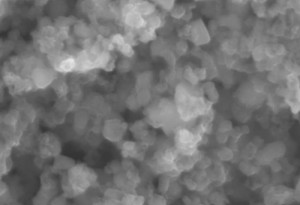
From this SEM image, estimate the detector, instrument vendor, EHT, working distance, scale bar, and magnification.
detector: SE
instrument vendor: Hitachi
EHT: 15 kV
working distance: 10.3 mm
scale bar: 500 nm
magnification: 60 K X